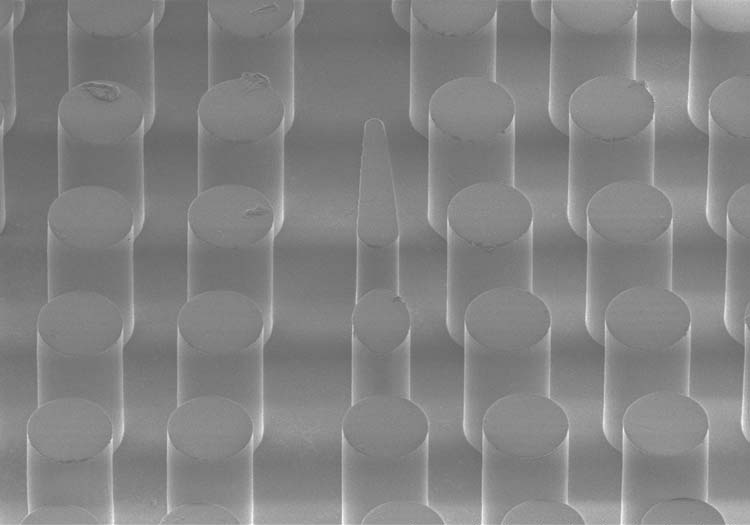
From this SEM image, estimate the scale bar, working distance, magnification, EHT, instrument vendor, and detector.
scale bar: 300000 nm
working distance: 15.2 mm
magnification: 0.18 K X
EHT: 0.9 kV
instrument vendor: Hitachi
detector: SE(M)